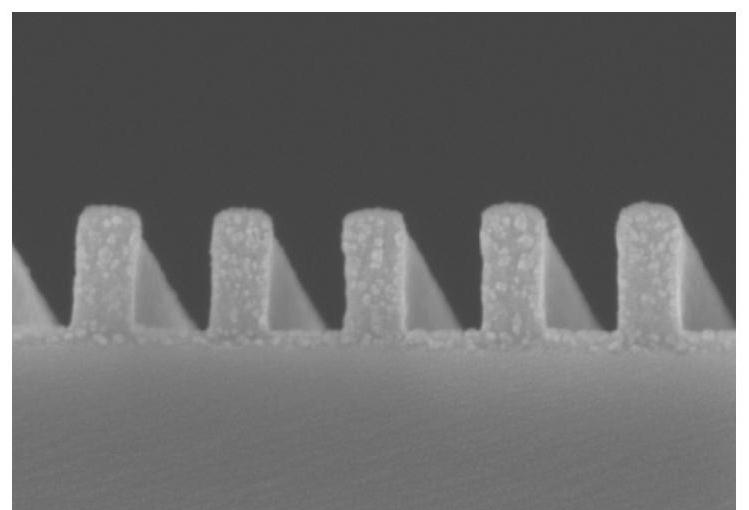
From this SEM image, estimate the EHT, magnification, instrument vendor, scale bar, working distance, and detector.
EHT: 5 kV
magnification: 70 K X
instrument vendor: Hitachi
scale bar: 500 nm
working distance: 13.7 mm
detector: SE(UL)